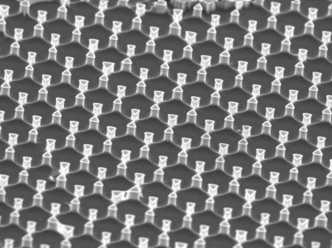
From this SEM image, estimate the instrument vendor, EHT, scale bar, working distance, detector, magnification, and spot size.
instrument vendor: FEI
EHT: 3 kV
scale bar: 5000 nm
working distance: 4.9 mm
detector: TLD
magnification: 10.864 K X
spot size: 2